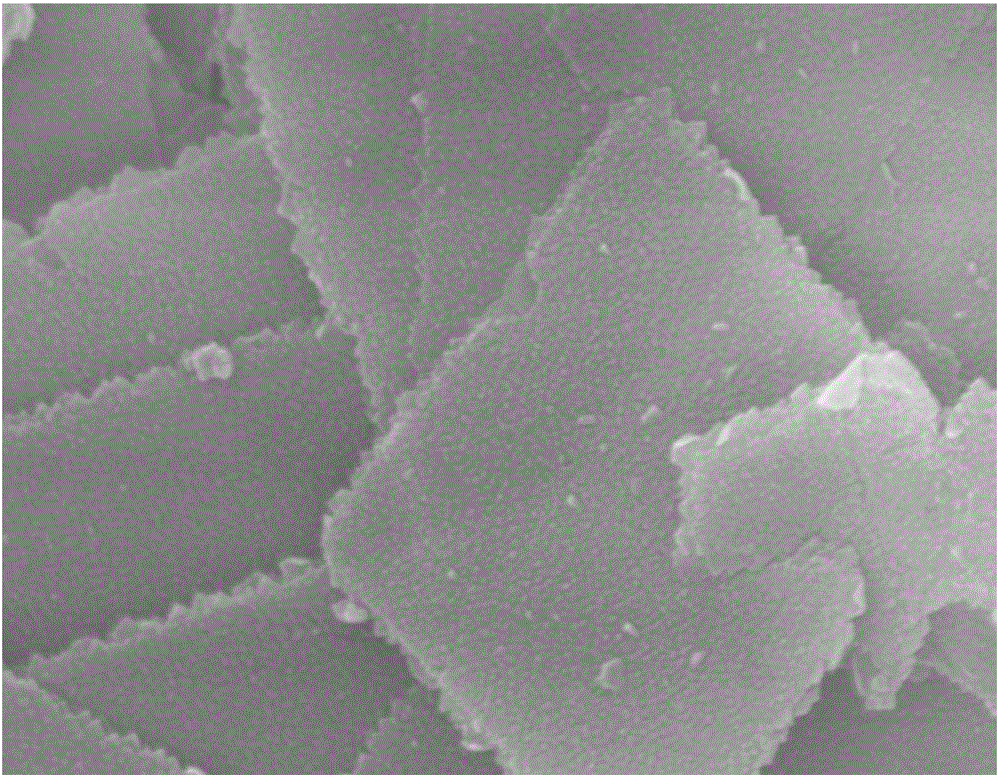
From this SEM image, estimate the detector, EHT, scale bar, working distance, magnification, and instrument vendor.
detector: SE(UL)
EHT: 15 kV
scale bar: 500 nm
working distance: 7.8 mm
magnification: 60 K X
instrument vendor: Hitachi